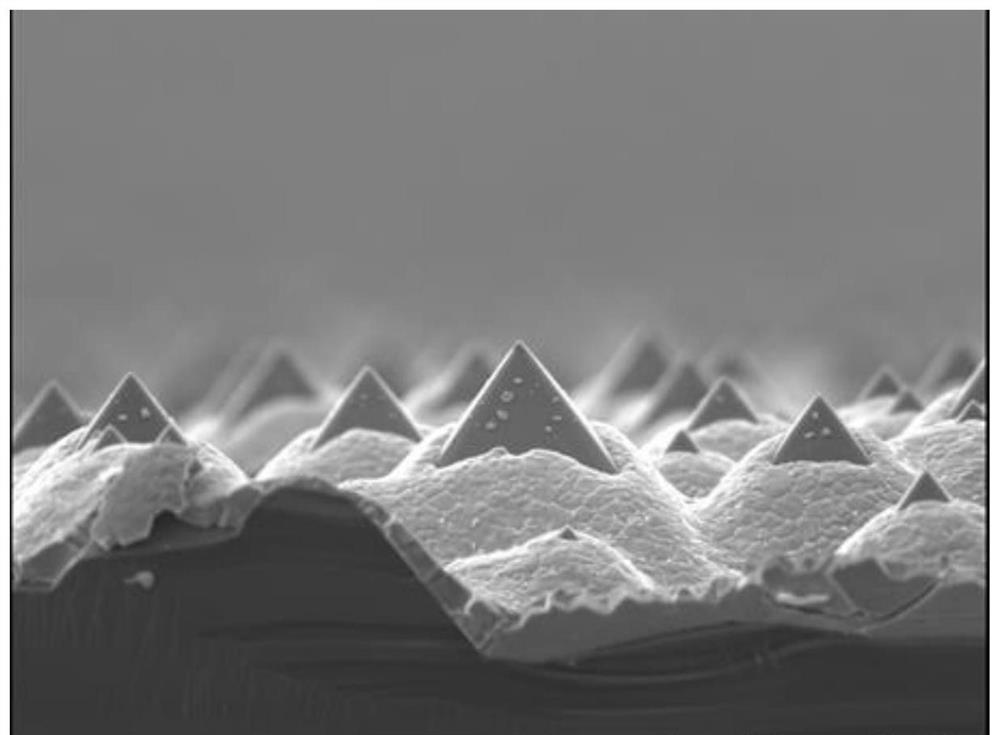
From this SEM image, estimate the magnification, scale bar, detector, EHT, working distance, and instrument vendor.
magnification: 5 K X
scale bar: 1000 nm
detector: LED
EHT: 3 kV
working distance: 15 mm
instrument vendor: JEOL